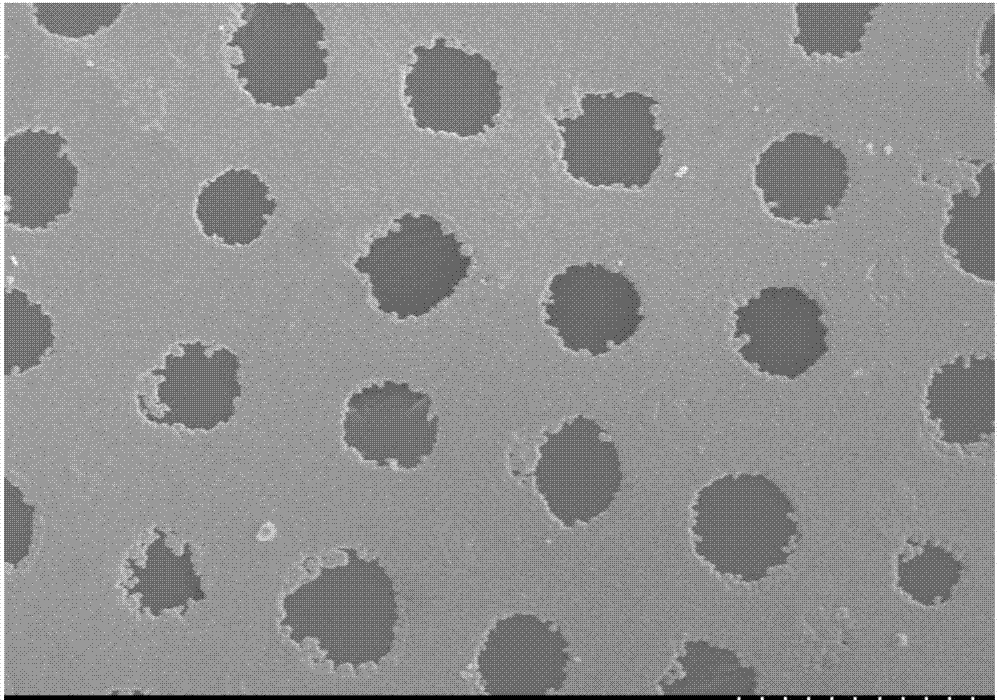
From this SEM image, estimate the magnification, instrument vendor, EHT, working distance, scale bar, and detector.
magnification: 0.3 K X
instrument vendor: Hitachi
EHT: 15 kV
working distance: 13 mm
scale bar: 100000 nm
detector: SE(M)